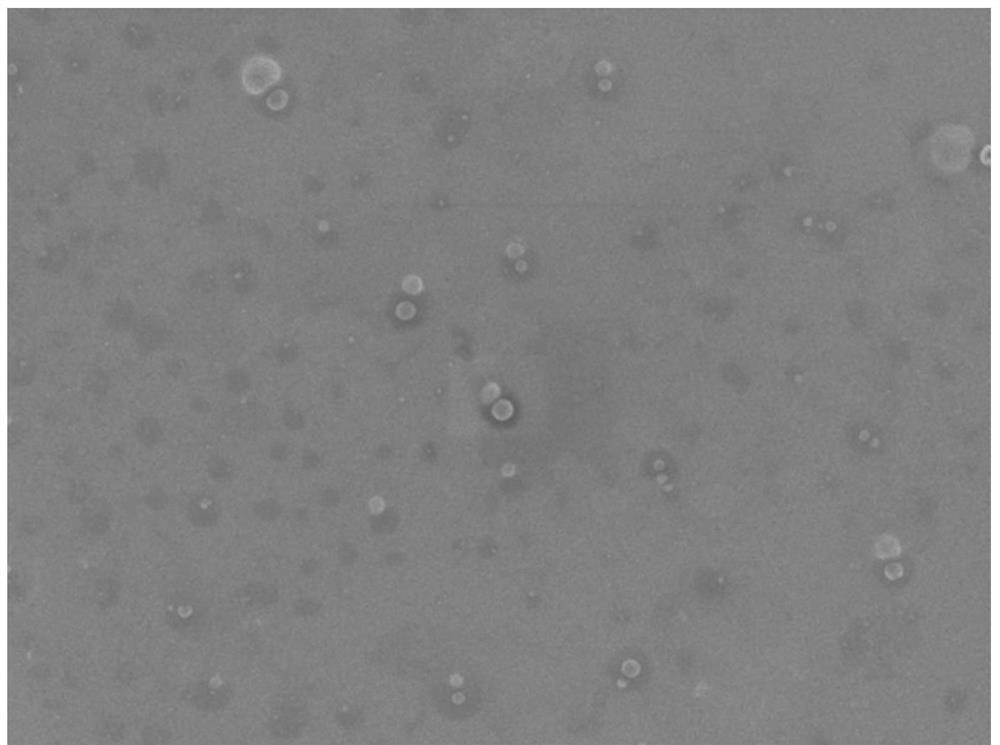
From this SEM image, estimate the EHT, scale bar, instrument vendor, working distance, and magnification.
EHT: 5 kV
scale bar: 1000 nm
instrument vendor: JEOL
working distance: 6.7 mm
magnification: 3 K X